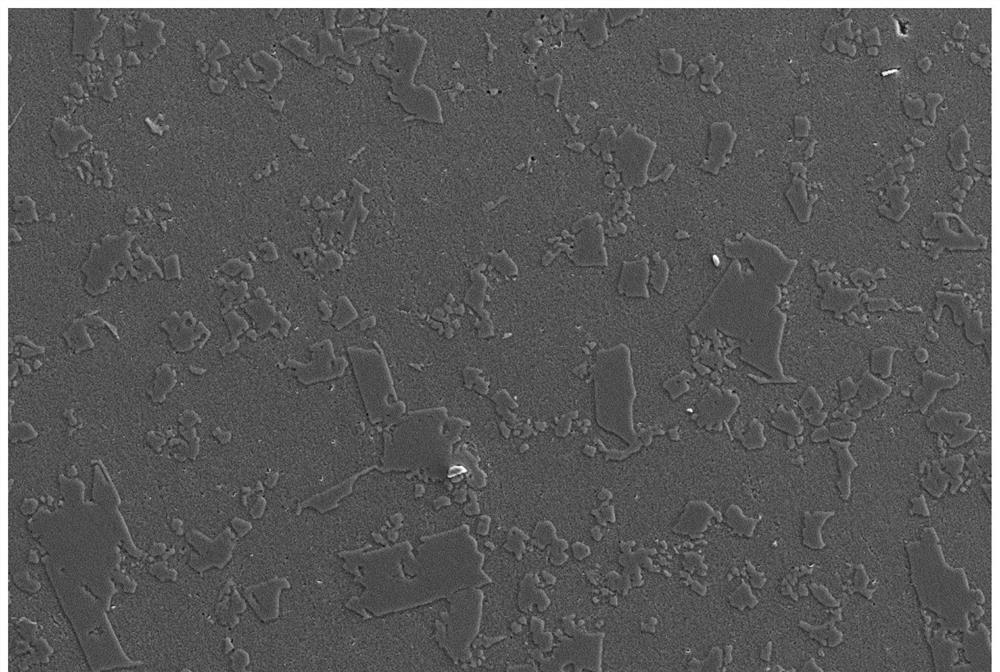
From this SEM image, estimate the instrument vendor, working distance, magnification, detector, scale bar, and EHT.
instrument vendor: Zeiss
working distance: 9.9 mm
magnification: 0.2 K X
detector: SE2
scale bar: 20000 nm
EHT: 20 kV